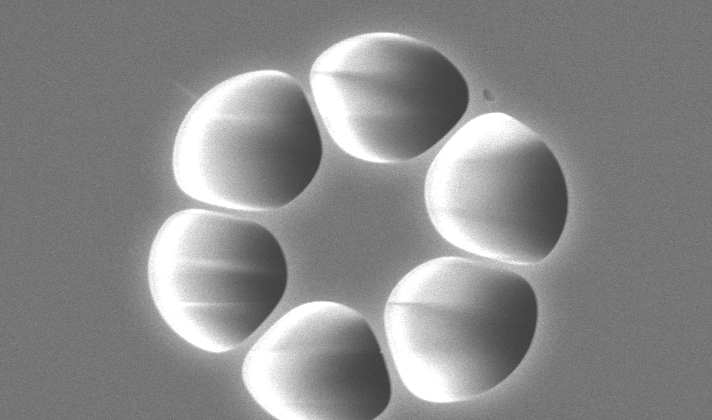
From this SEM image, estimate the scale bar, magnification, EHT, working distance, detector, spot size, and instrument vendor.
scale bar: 10000 nm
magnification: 2 K X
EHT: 20 kV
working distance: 12.4 mm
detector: SE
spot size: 4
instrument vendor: FEI